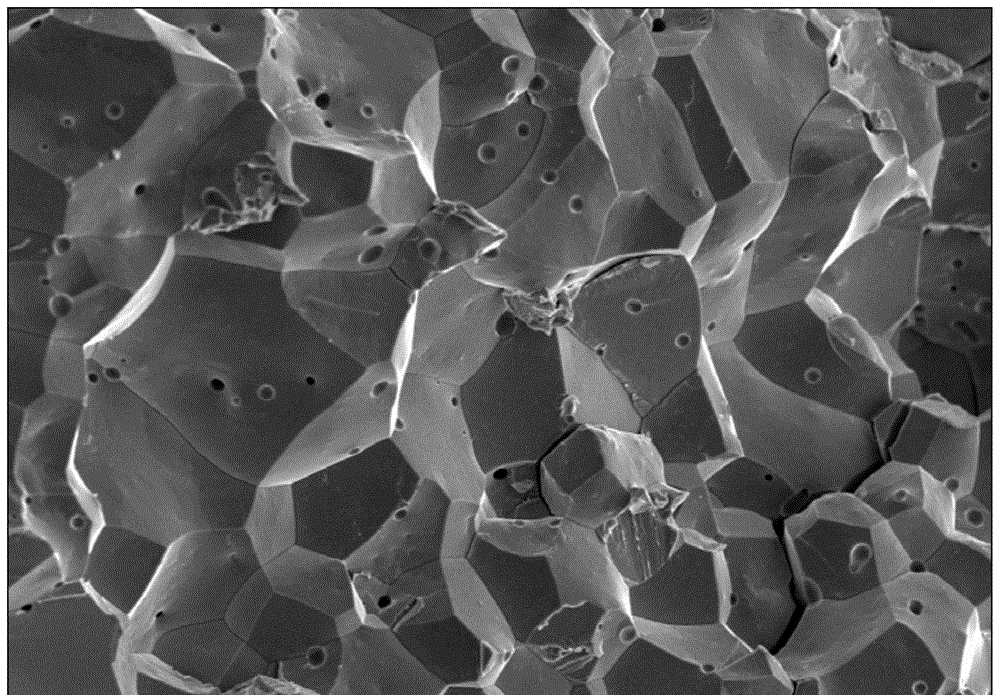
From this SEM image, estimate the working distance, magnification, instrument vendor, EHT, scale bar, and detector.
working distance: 13.3 mm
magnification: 0.5 K X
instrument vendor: Hitachi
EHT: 20 kV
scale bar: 100000 nm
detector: SE